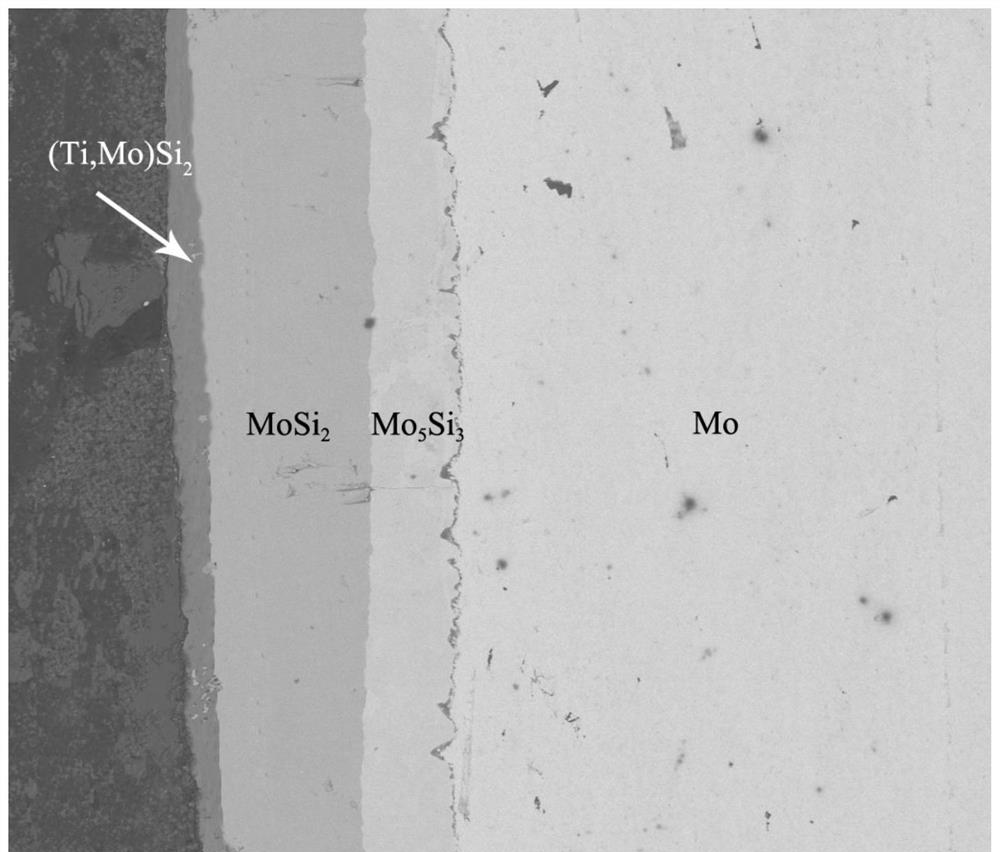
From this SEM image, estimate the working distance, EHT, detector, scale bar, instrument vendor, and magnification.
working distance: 15.9 mm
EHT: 10 kV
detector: BSED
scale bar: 100000 nm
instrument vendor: FEI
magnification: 1.5 K X